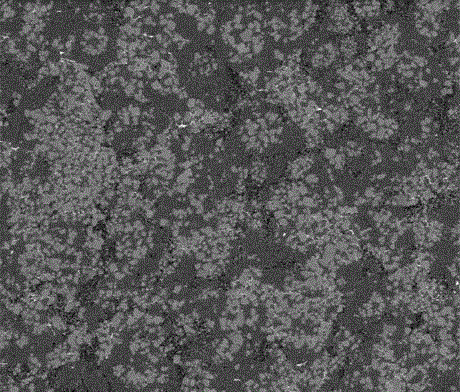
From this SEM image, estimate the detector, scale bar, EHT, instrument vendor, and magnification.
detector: BSED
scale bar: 100000 nm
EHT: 8 kV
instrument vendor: FEI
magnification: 0.5 K X